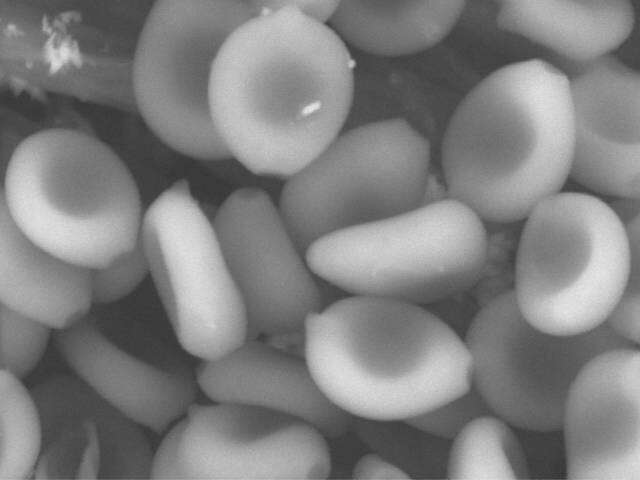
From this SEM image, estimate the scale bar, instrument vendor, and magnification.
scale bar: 10000 nm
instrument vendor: Hitachi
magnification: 10 K X